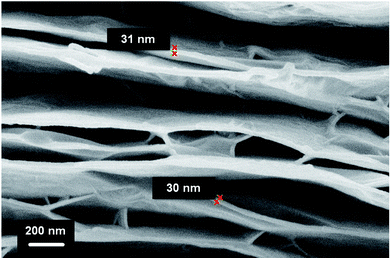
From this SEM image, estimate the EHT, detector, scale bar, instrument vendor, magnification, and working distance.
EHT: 2.7 kV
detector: InLens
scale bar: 200 nm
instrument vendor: Zeiss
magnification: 50 K X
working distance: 7 mm